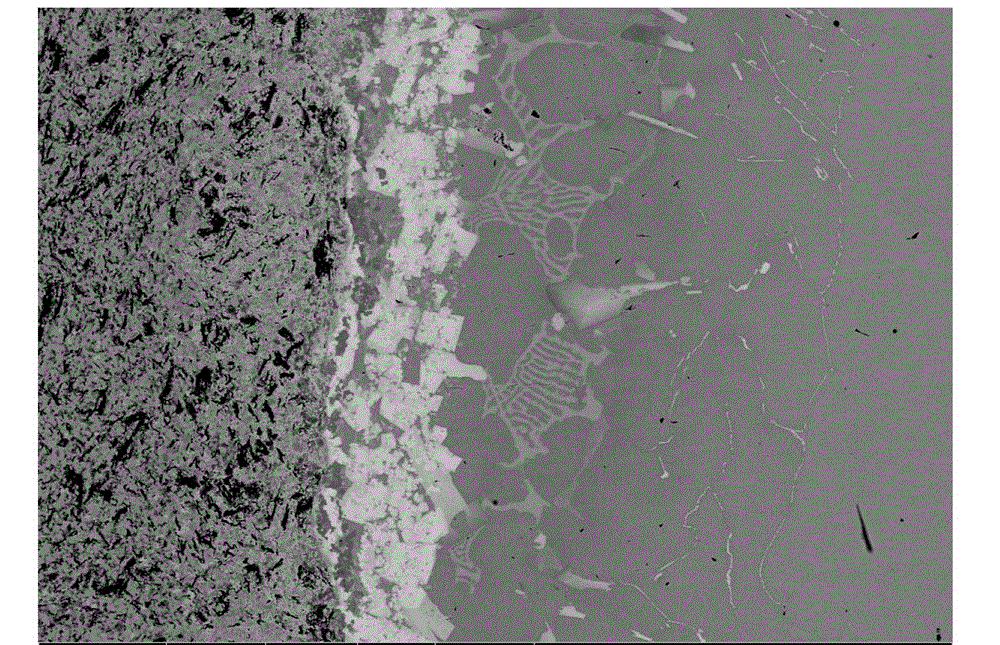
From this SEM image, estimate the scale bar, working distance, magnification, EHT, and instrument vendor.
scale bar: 200000 nm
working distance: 10.4 mm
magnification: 0.5 K X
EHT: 20 kV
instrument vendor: FEI